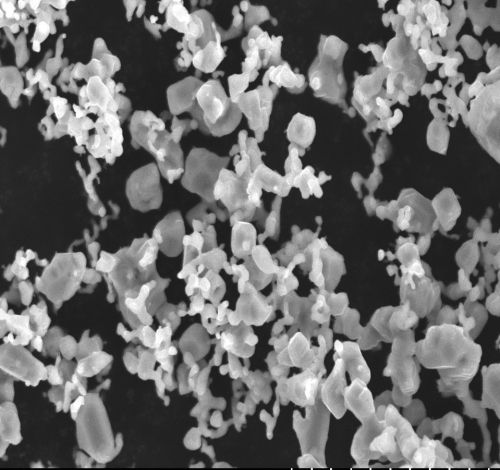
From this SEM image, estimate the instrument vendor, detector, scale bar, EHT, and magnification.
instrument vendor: JEOL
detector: SE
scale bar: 10000 nm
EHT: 15 kV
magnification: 5 K X